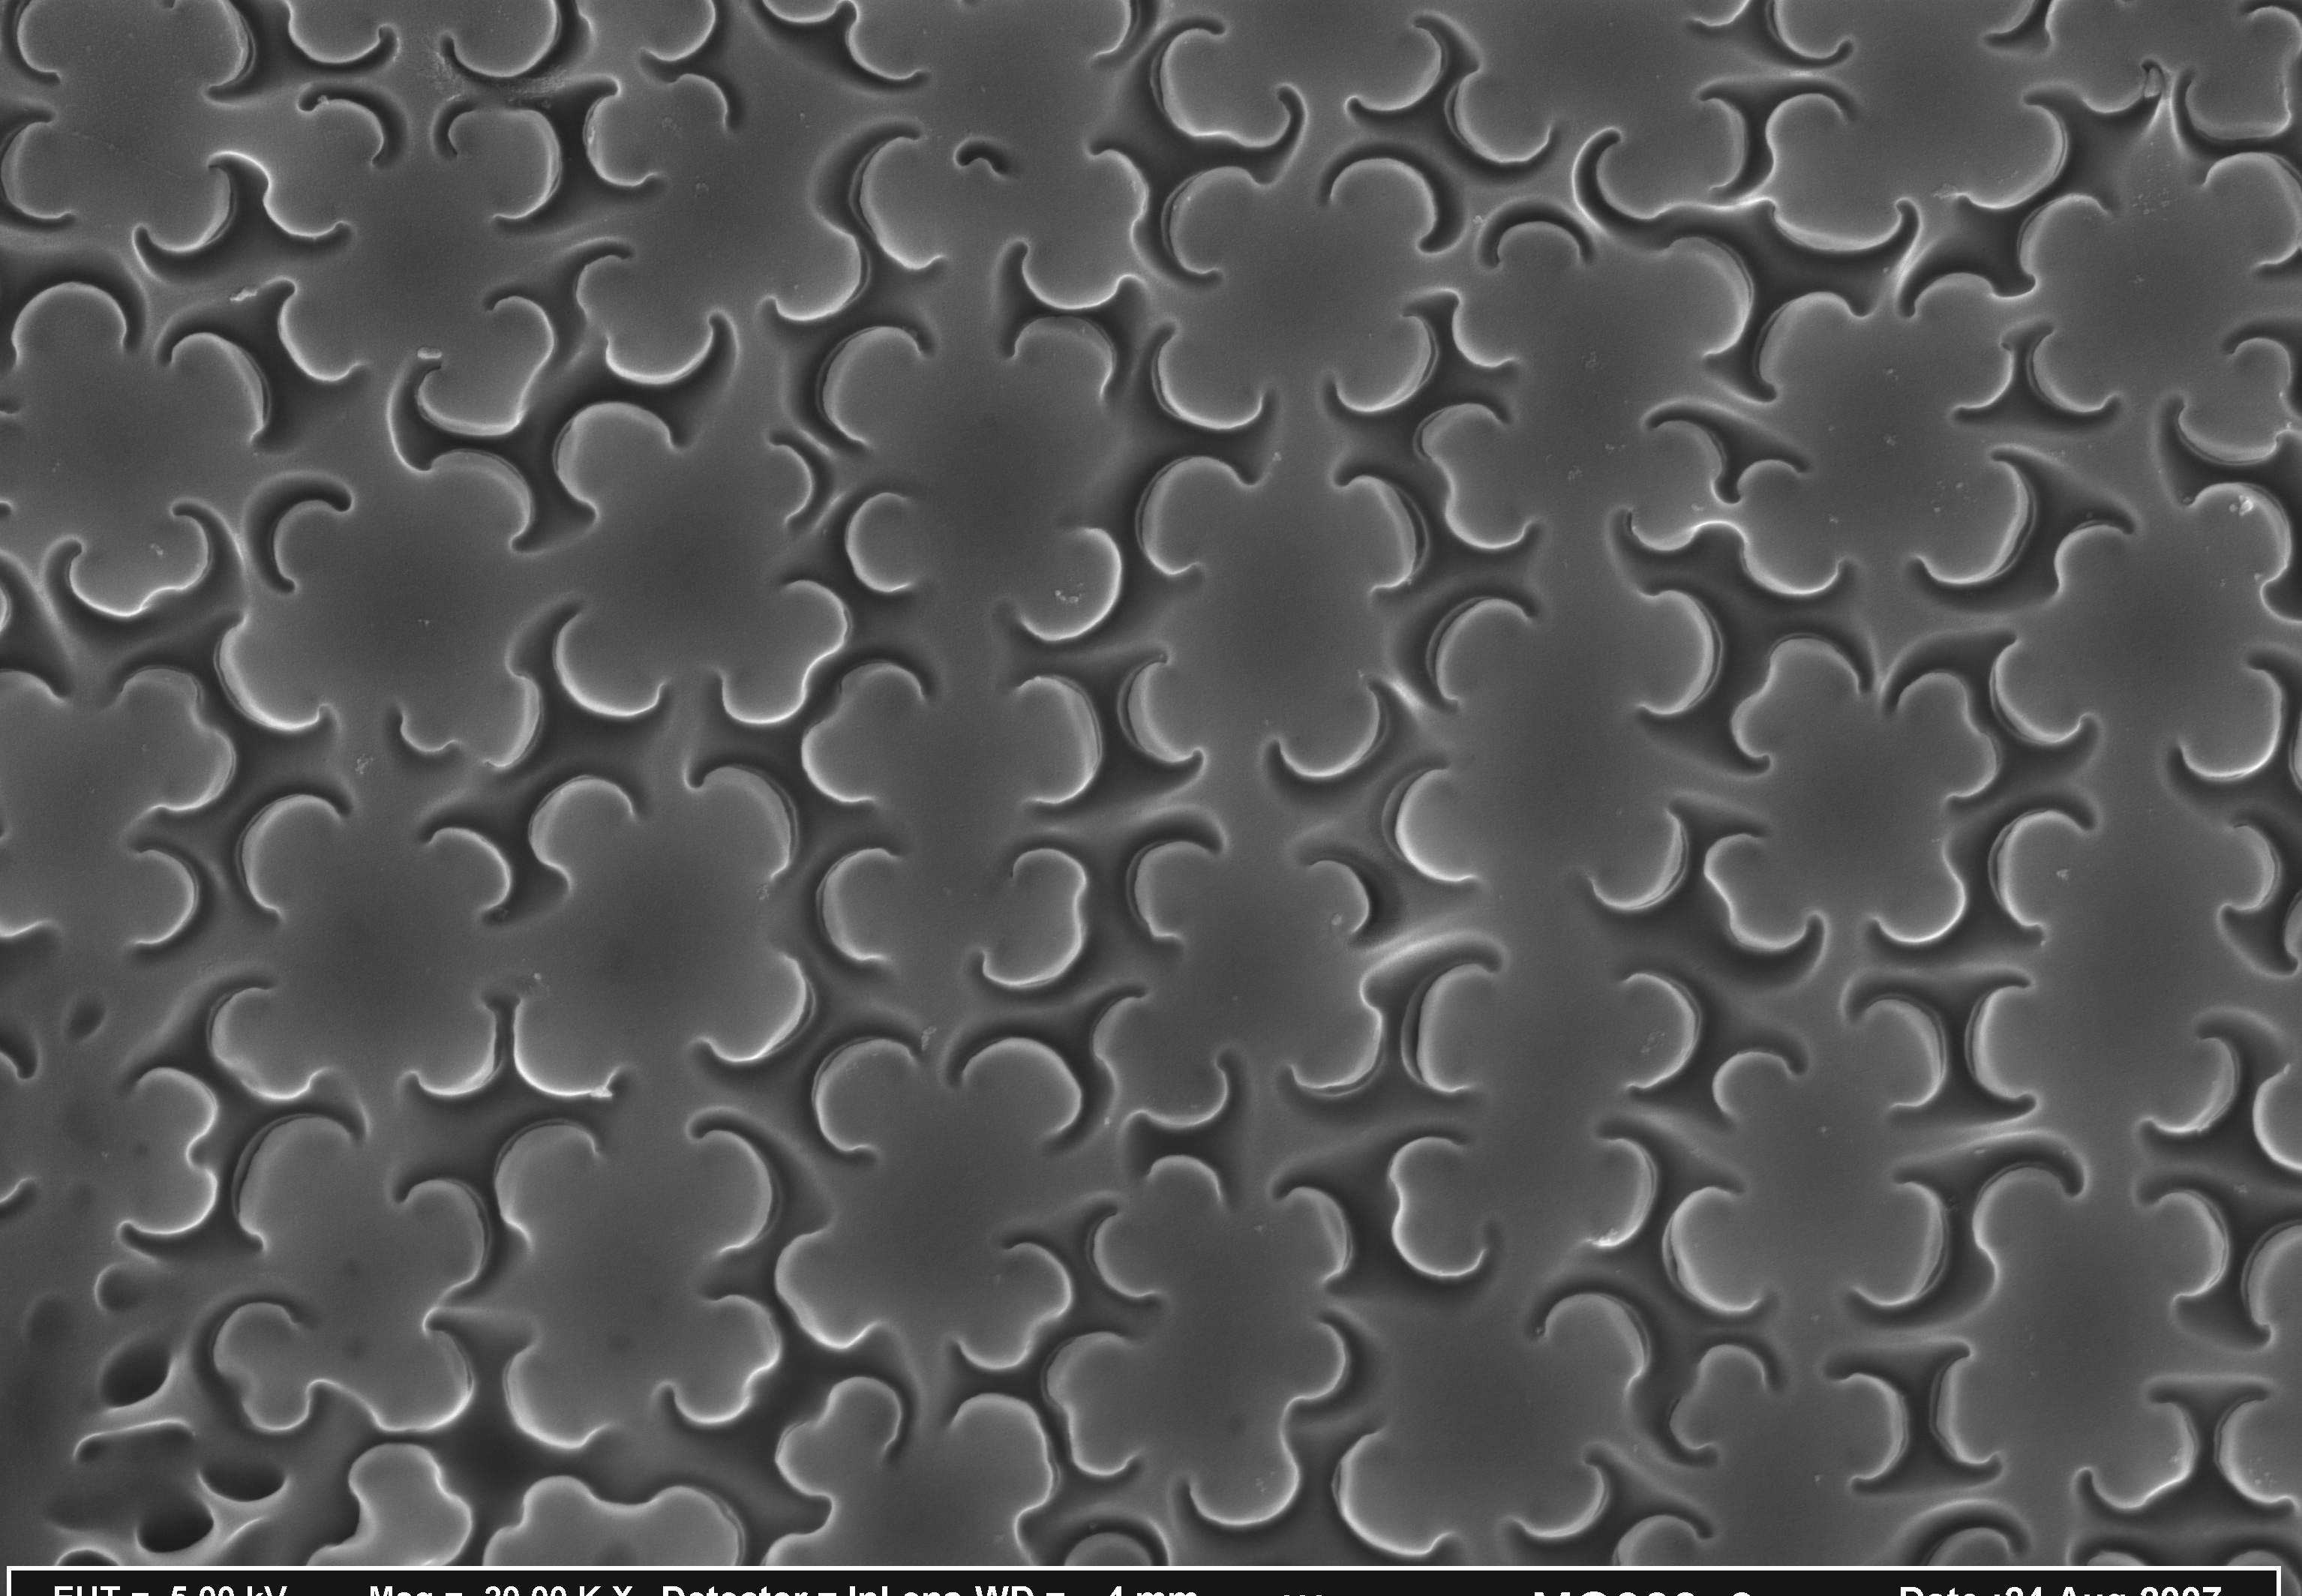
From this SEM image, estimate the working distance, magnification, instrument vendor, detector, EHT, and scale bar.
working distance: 4 mm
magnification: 20 K X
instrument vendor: Zeiss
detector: InLens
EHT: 5 kV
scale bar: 200 nm